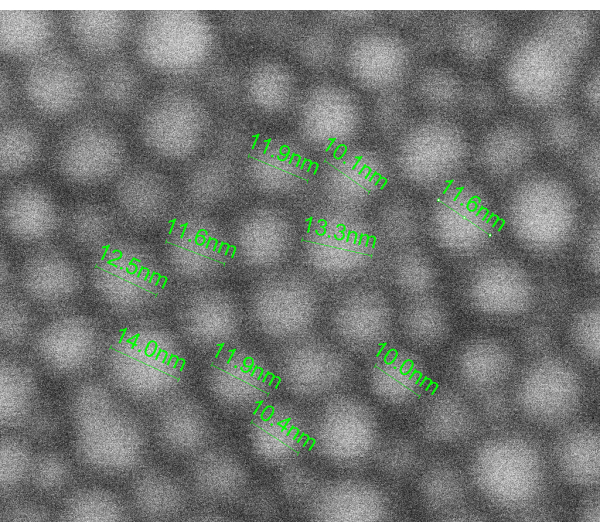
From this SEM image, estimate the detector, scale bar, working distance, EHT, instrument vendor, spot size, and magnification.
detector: SE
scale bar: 10 nm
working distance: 5.4 mm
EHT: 10 kV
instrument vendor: FEI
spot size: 2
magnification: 2677.71 K X